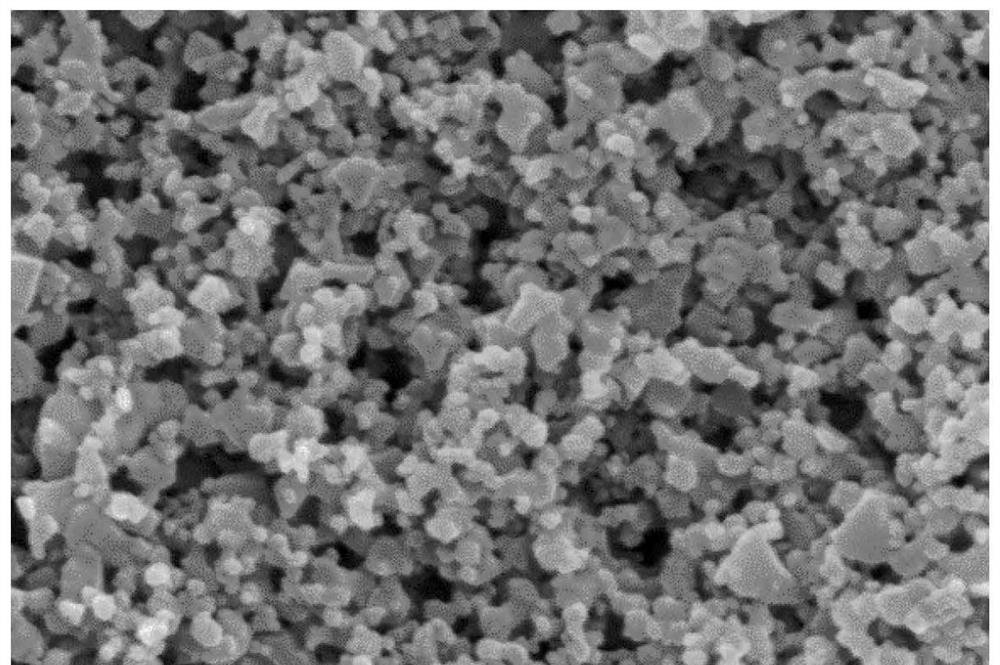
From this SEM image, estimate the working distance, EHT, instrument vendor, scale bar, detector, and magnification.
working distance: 8.2 mm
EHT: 5 kV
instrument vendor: Zeiss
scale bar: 200 nm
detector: InLens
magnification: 50 K X